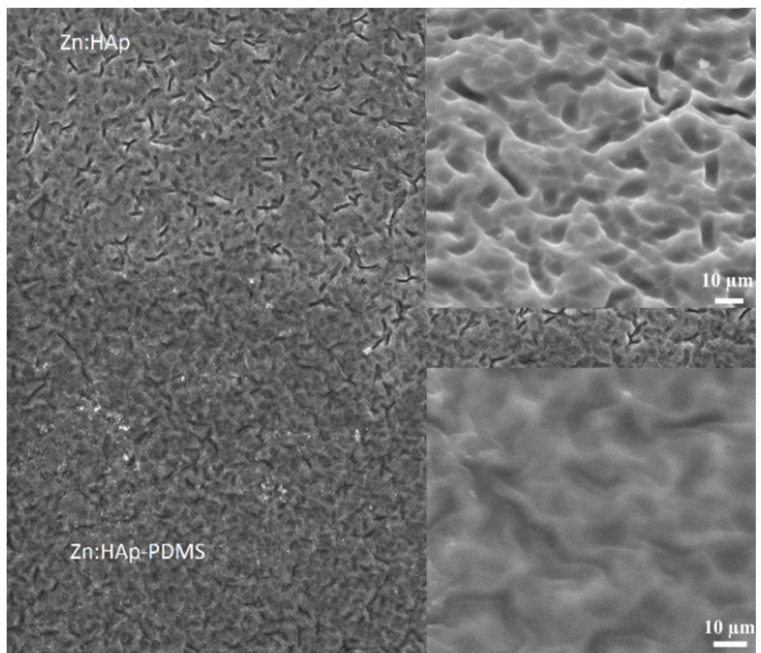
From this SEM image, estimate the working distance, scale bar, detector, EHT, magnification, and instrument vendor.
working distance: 9.8 mm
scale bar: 300000 nm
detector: ETD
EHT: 30 kV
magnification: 0.5 K X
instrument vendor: FEI